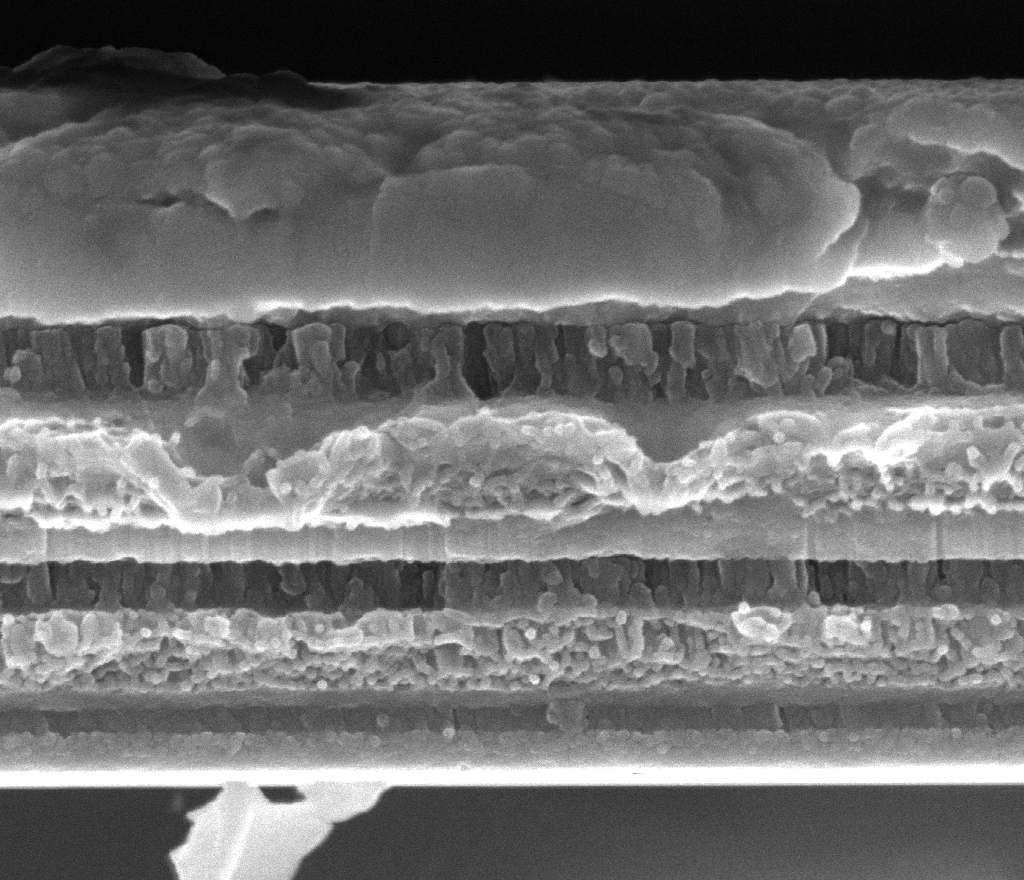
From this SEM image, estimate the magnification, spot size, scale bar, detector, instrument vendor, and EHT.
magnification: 20 K X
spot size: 3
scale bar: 2000 nm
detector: TLD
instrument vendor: FEI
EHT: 15 kV